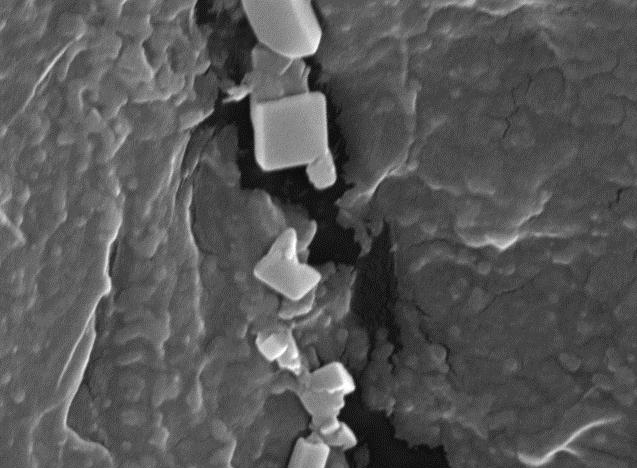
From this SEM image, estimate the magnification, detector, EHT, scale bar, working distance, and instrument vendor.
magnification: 30 K X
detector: SEI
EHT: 15 kV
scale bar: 100 nm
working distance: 13 mm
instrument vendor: JEOL